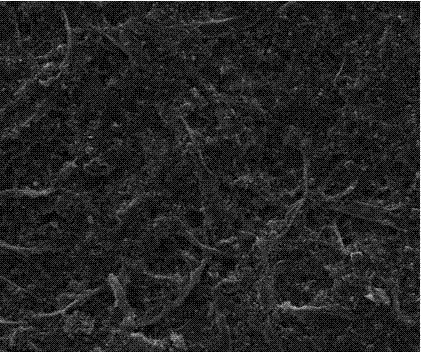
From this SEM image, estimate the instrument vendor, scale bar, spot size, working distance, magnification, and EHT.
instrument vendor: FEI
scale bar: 200000 nm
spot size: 2.5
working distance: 8.8 mm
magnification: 0.4 K X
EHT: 20 kV